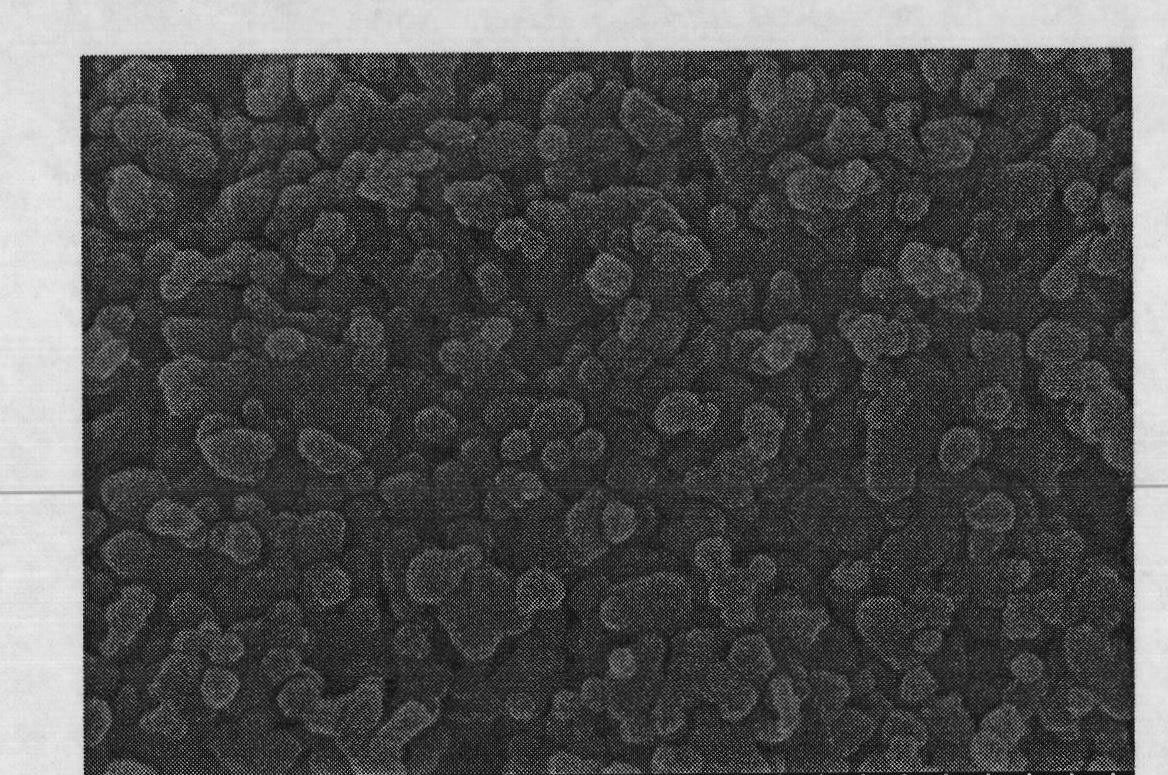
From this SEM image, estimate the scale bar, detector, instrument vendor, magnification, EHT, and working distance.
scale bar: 500 nm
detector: SE(U)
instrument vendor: Hitachi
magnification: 100 K X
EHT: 8 kV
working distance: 4 mm